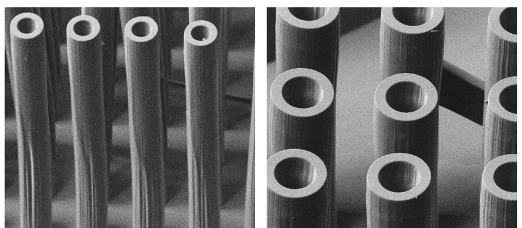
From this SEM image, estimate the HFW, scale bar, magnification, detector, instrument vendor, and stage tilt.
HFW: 1230 µm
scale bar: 200000 nm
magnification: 0.247 K X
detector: CDM-I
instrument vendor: FEI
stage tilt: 45.4°